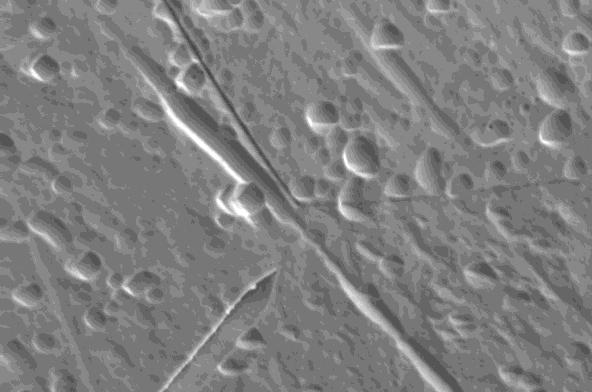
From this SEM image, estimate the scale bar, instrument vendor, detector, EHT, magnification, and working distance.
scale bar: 10000 nm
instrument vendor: Zeiss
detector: SE1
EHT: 15 kV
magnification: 3 K X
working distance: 8 mm